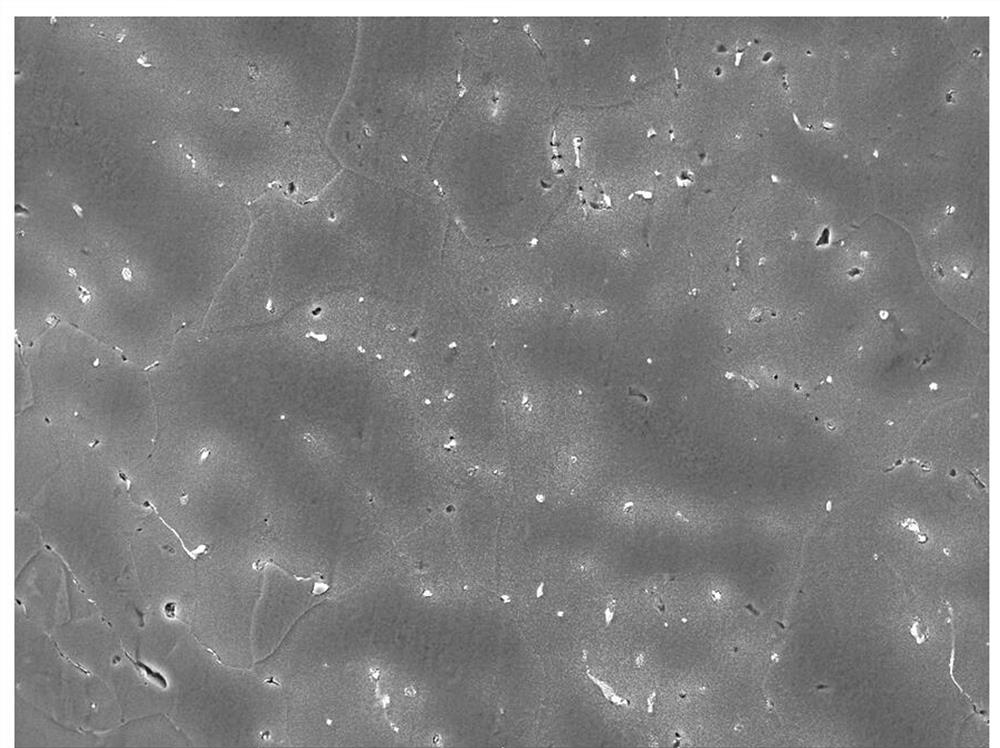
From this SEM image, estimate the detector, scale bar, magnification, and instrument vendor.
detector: SE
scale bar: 50000 nm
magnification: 1 K X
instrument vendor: Tescan